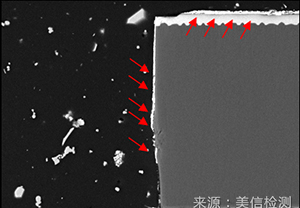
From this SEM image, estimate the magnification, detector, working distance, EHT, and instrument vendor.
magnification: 0.8 K X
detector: BSE3D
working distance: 10.1 mm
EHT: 15 kV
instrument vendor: Hitachi